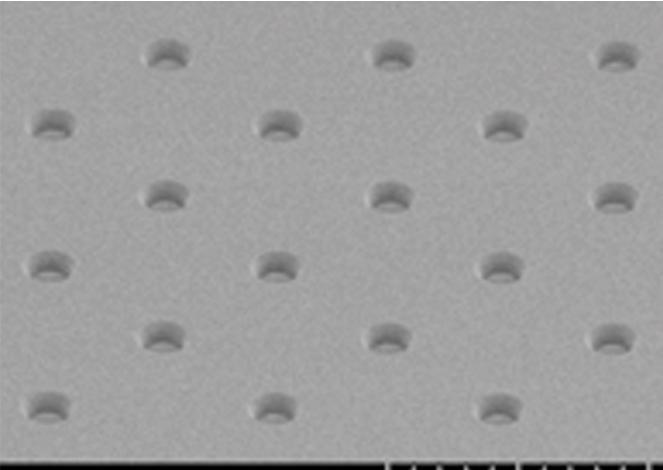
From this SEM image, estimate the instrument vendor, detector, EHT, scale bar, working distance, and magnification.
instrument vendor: Hitachi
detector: SE(UL)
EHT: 1 kV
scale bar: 5000 nm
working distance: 6 mm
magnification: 10 K X